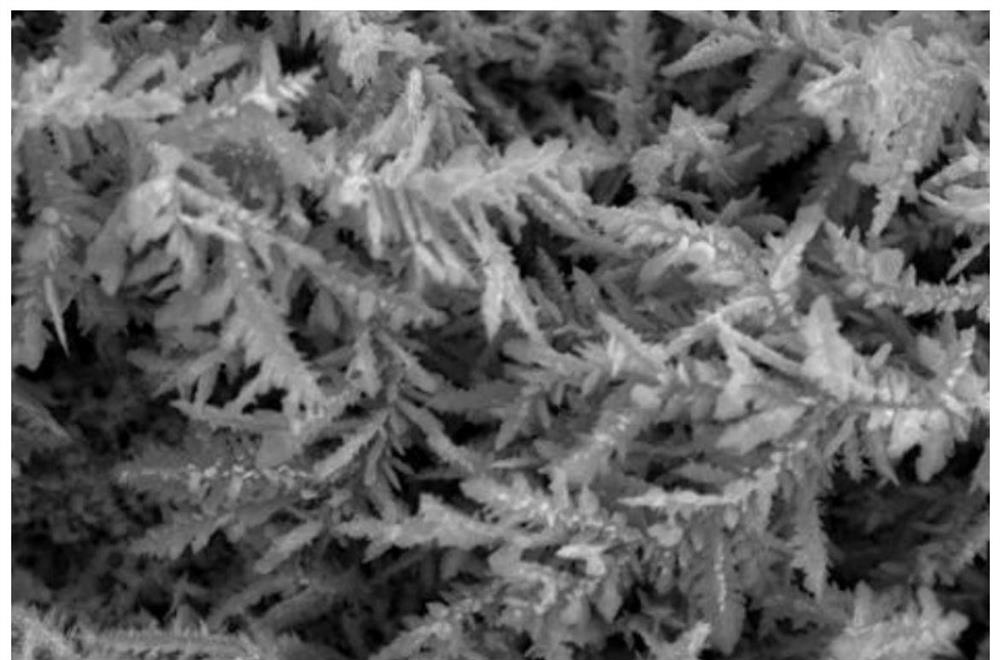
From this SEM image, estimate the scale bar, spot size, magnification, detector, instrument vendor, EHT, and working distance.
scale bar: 10000 nm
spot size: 30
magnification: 1 K X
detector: SEI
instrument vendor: JEOL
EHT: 30 kV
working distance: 11 mm